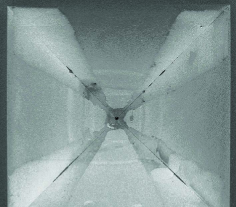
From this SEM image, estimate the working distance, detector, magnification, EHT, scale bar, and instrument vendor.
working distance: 16.9 mm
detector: SEI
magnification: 0.065 K X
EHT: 10 kV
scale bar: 100000 nm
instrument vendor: JEOL